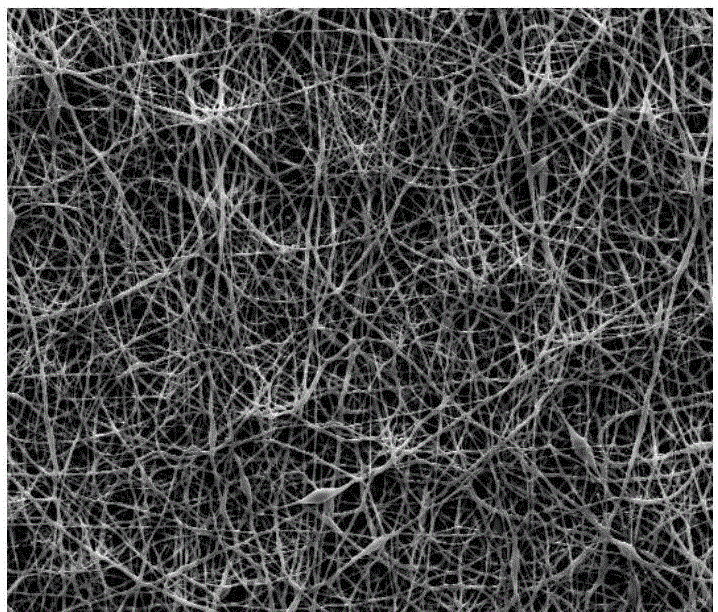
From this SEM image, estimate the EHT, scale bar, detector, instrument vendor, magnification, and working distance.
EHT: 20 kV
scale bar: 100000 nm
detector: SE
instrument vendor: FEI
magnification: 1 K X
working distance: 11.9 mm